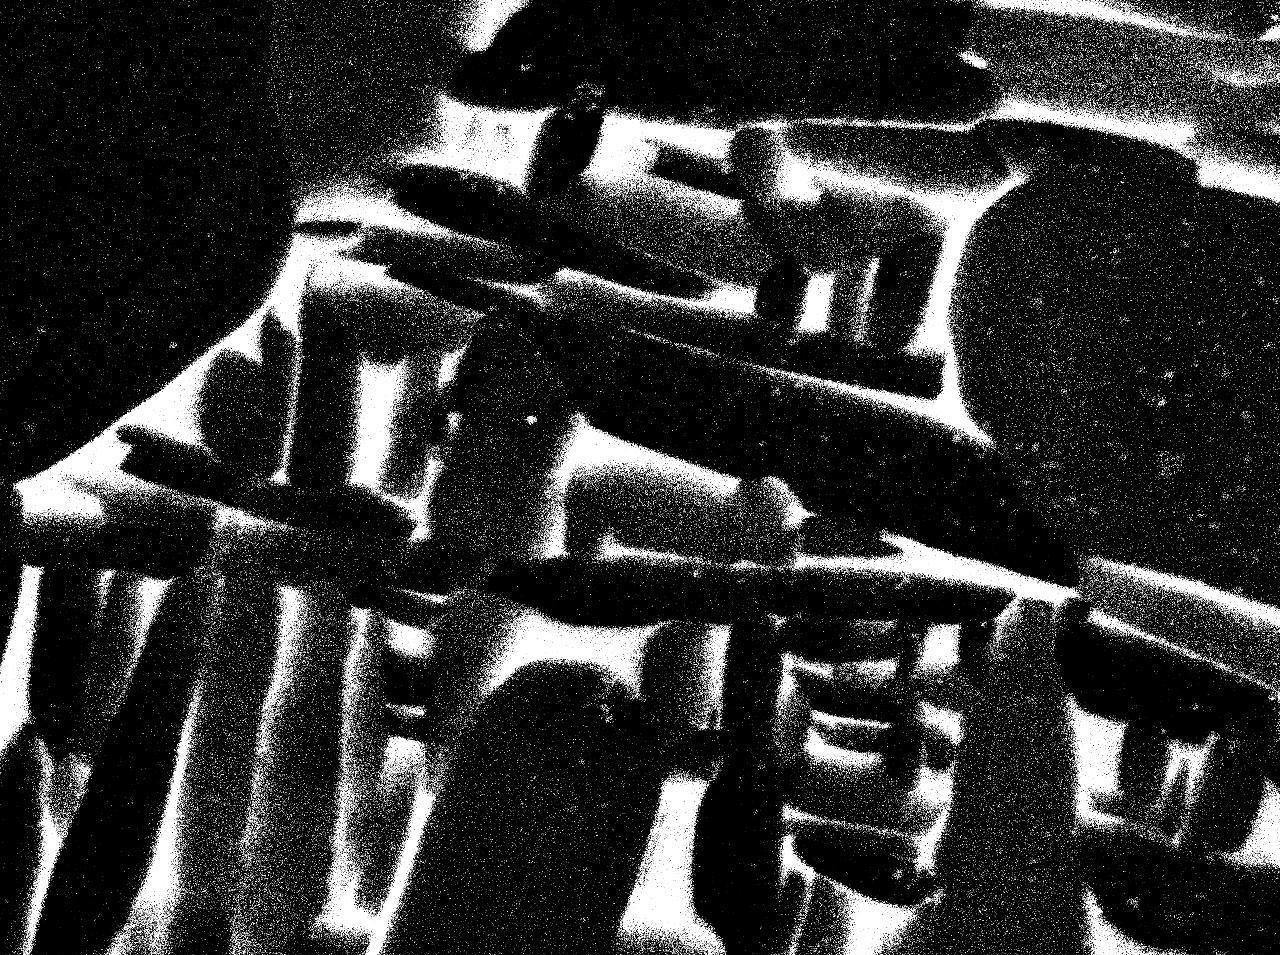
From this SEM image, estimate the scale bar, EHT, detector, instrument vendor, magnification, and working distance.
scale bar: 1000 nm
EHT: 15 kV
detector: COMPO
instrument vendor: JEOL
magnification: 10 K X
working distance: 10.1 mm